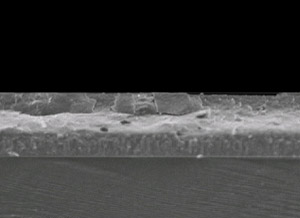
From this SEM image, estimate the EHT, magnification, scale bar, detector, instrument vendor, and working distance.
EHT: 3 kV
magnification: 8.5 K X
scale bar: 1000 nm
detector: SEI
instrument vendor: JEOL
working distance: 2.9 mm